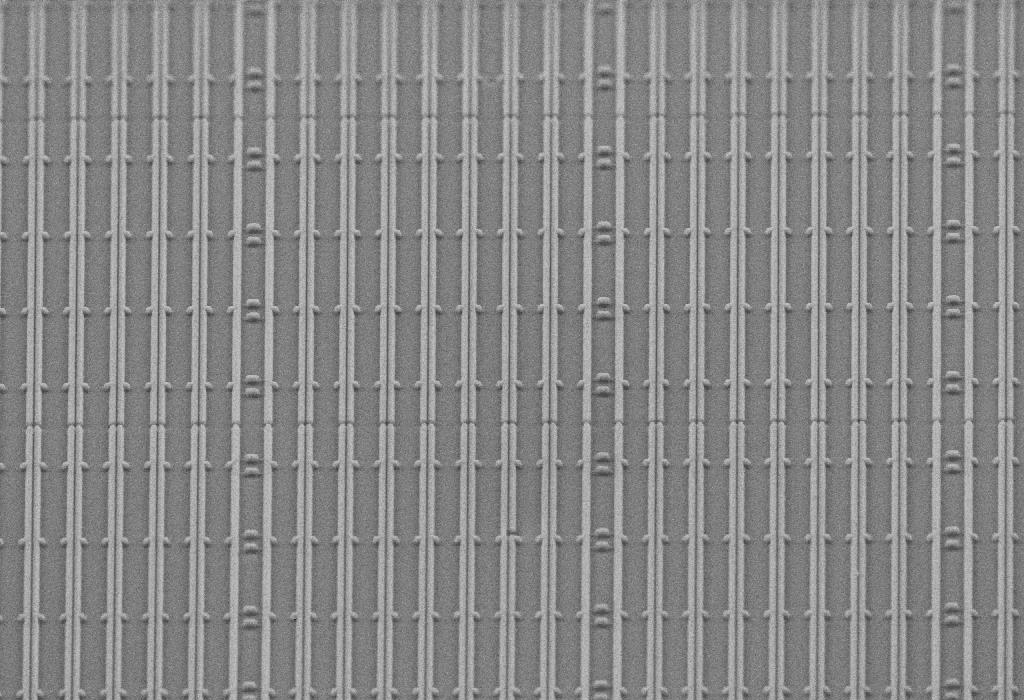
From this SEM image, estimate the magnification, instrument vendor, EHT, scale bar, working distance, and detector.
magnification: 3 K X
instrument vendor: Zeiss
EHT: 10 kV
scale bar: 2000 nm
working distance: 8 mm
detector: SE2